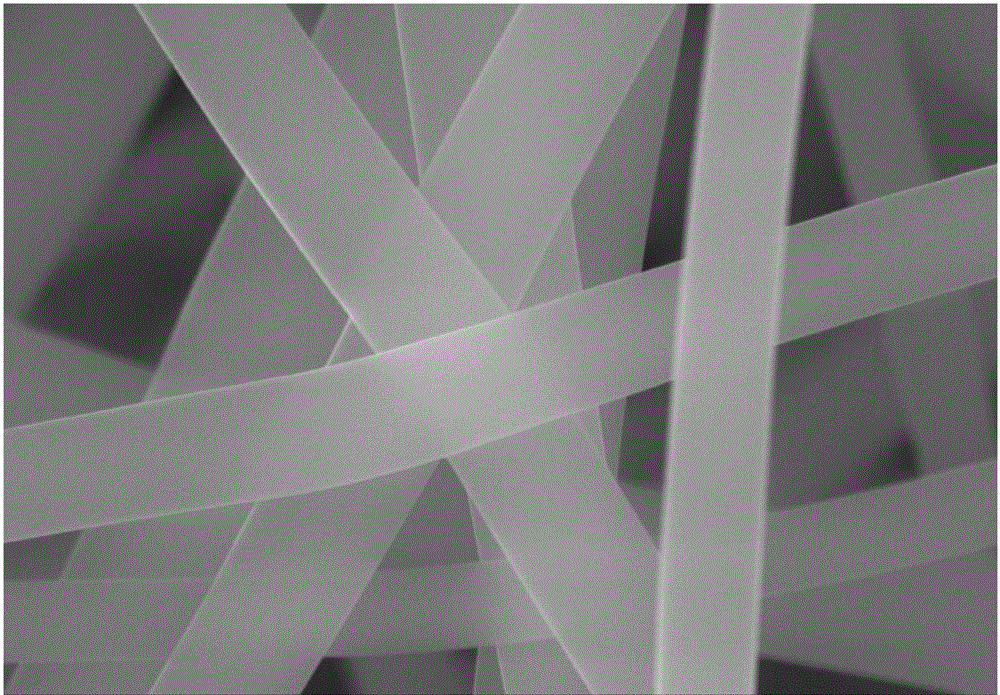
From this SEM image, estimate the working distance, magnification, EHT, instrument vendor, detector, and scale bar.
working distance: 5.7 mm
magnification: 100 K X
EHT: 20 kV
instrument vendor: Zeiss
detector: InLens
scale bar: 200 nm